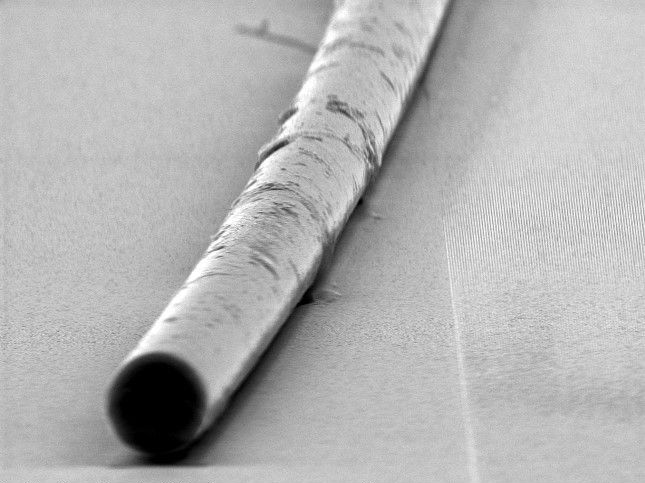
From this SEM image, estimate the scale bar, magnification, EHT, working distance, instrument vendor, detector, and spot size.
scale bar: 5000 nm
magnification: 5.607 K X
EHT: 10 kV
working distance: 9.6 mm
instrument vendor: FEI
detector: SE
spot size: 3